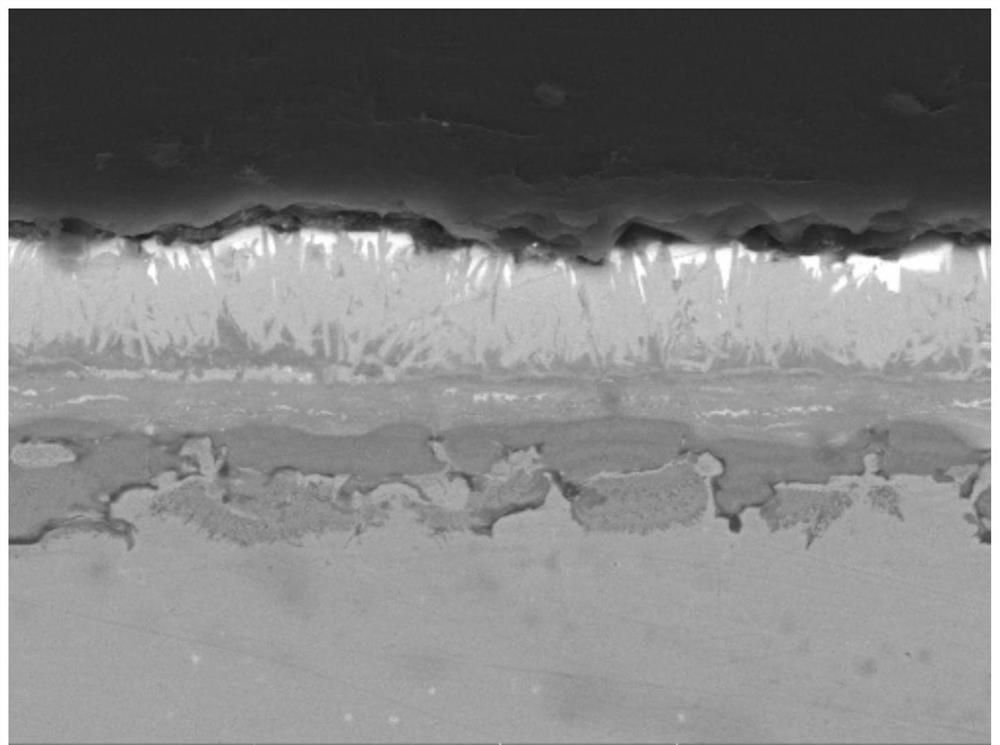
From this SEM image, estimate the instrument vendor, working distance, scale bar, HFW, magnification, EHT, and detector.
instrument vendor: Tescan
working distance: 14.59 mm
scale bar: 10000 nm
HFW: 55.4 µm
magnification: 5 K X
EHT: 15 kV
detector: BSE